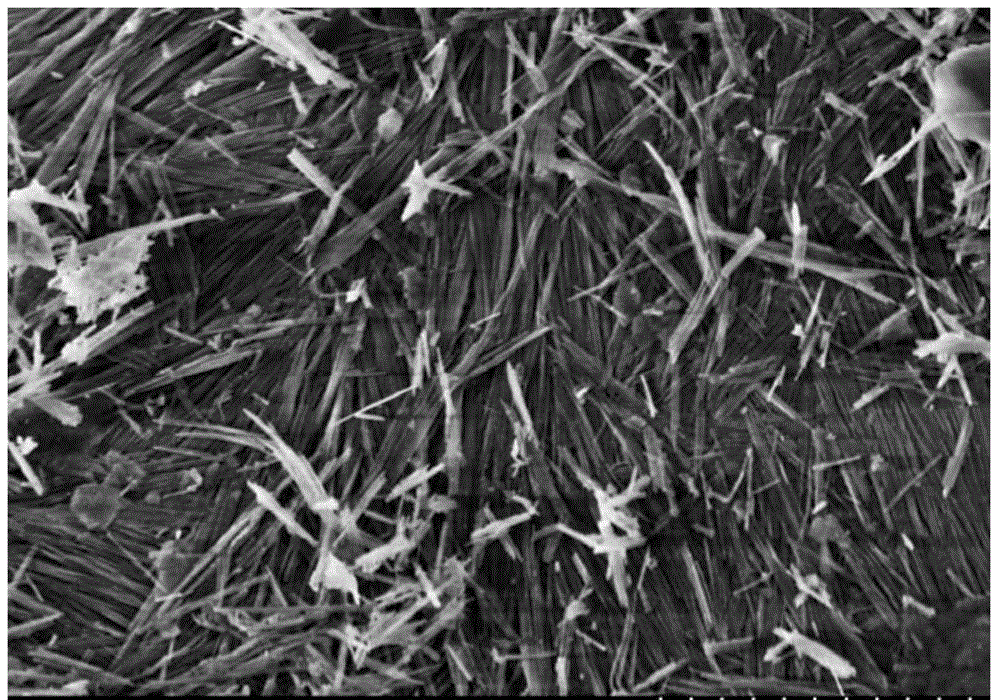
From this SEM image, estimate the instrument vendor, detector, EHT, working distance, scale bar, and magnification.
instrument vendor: Hitachi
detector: SE(M)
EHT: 2 kV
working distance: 4.3 mm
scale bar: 2000 nm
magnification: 20 K X